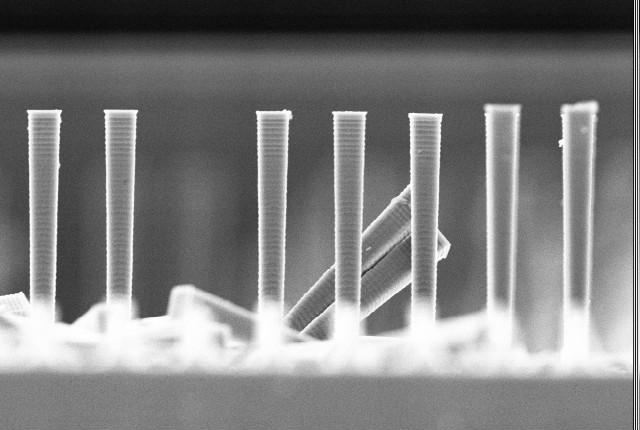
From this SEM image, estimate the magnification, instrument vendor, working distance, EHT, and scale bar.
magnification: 1.5 K X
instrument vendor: JEOL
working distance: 47 mm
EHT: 10 kV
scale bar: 10000 nm